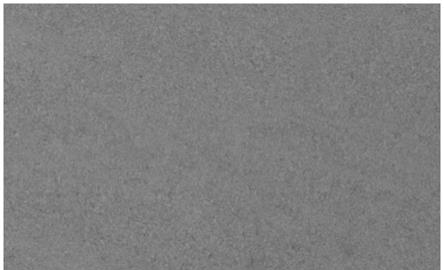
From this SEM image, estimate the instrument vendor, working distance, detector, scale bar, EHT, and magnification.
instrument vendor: Zeiss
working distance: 5.1 mm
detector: SE2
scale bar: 10000 nm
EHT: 15 kV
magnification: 1 K X